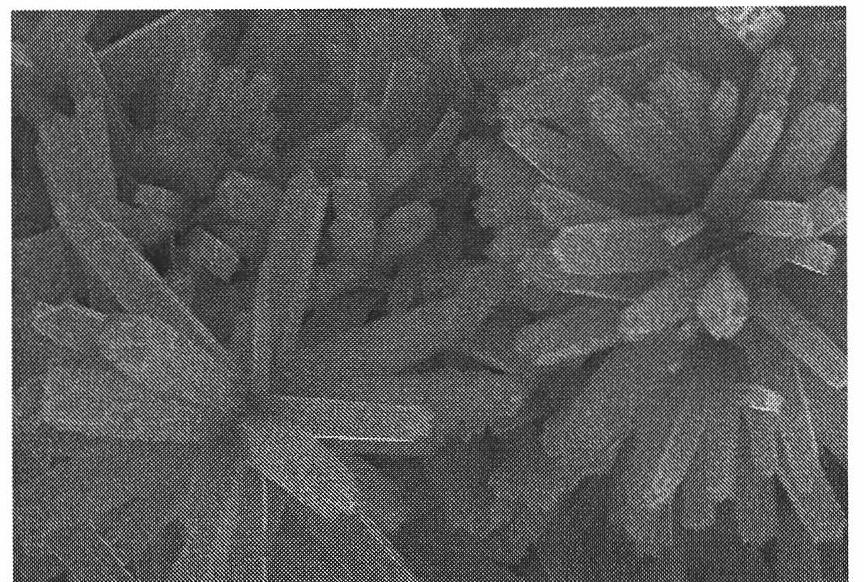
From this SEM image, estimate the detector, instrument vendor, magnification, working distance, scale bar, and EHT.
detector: SE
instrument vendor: Hitachi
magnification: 10 K X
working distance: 7.4 mm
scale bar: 5000 nm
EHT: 15 kV